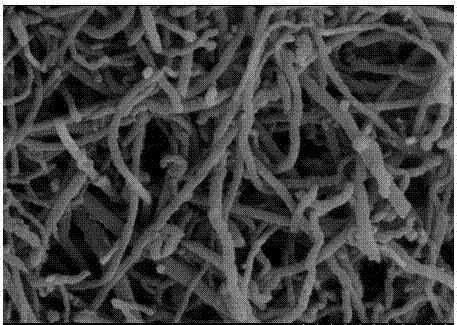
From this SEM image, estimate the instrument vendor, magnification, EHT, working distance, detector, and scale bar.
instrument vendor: Hitachi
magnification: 80 K X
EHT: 3 kV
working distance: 11.1 mm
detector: SE(M)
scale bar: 500 nm